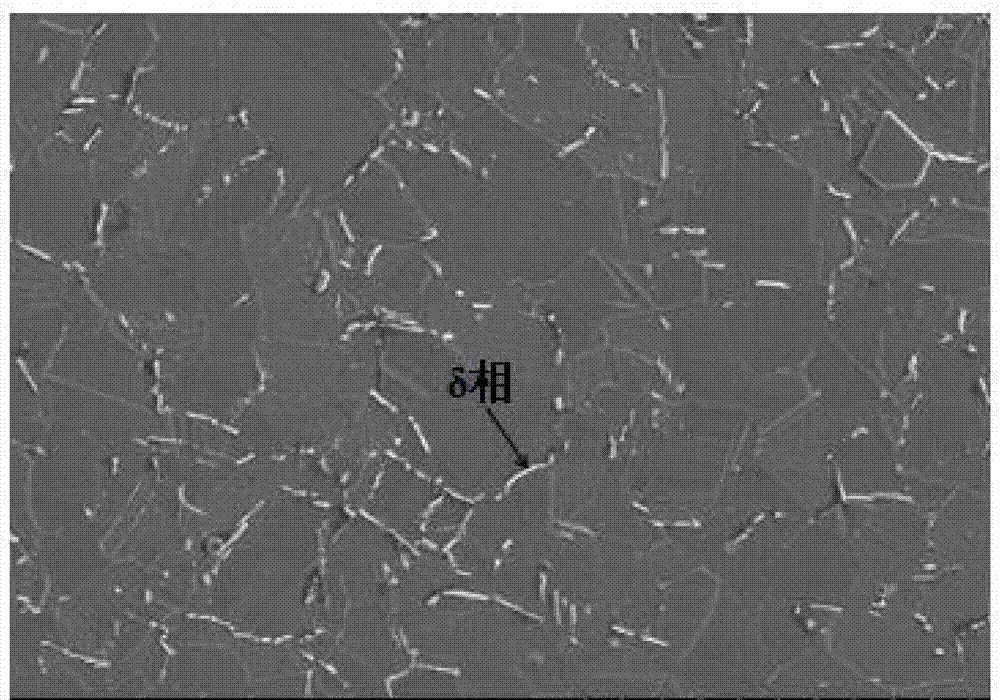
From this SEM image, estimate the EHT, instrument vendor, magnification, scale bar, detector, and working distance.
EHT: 15 kV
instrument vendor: Hitachi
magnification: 1 K X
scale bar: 50000 nm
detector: SE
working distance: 9.5 mm